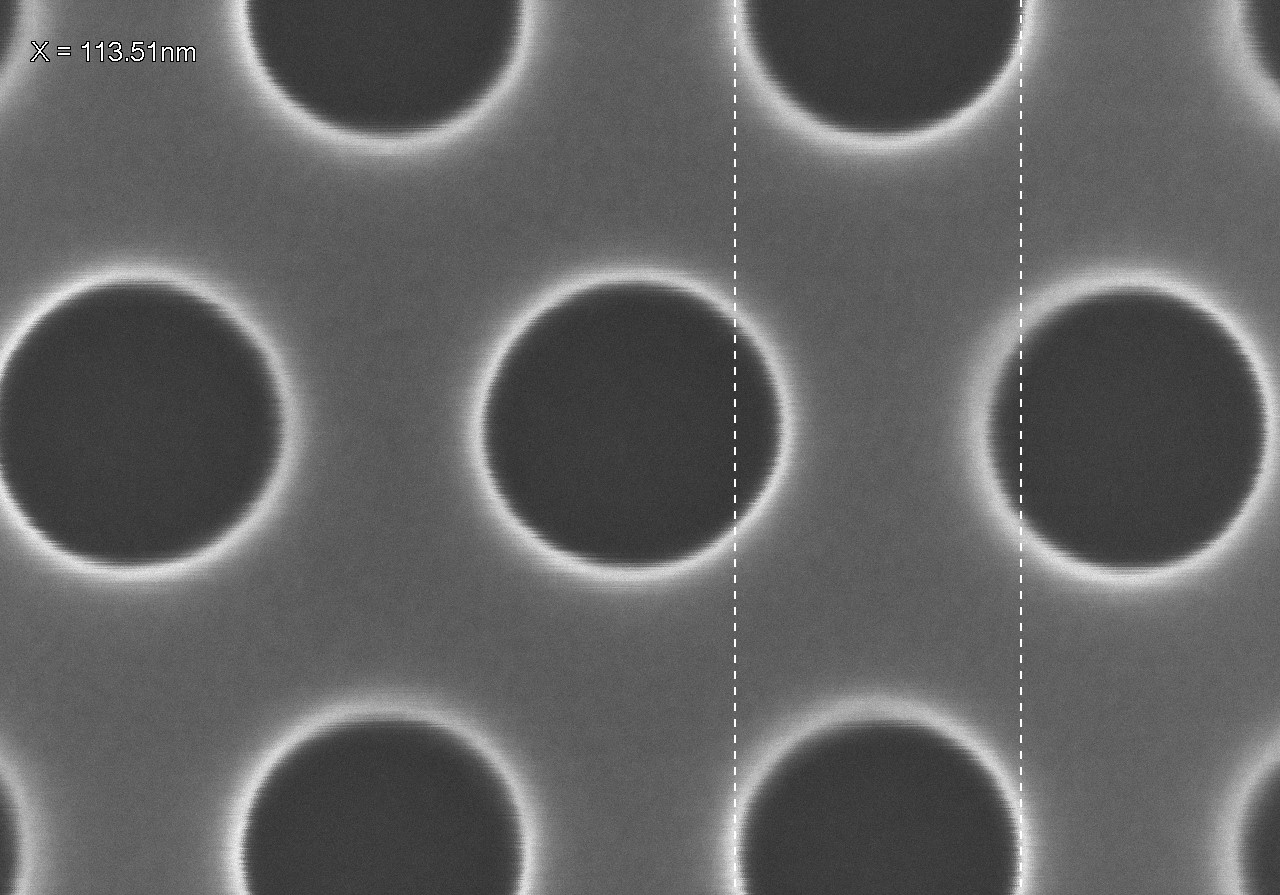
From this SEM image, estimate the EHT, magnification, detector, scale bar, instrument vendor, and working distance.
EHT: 5 kV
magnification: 250 K X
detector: SE(U)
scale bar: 200 nm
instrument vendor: Hitachi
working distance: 8.1 mm